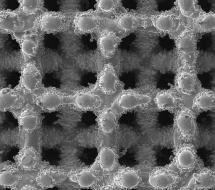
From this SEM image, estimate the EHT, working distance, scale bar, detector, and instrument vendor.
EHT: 20 kV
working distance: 25.53 mm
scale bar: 500000 nm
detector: SE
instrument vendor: Tescan